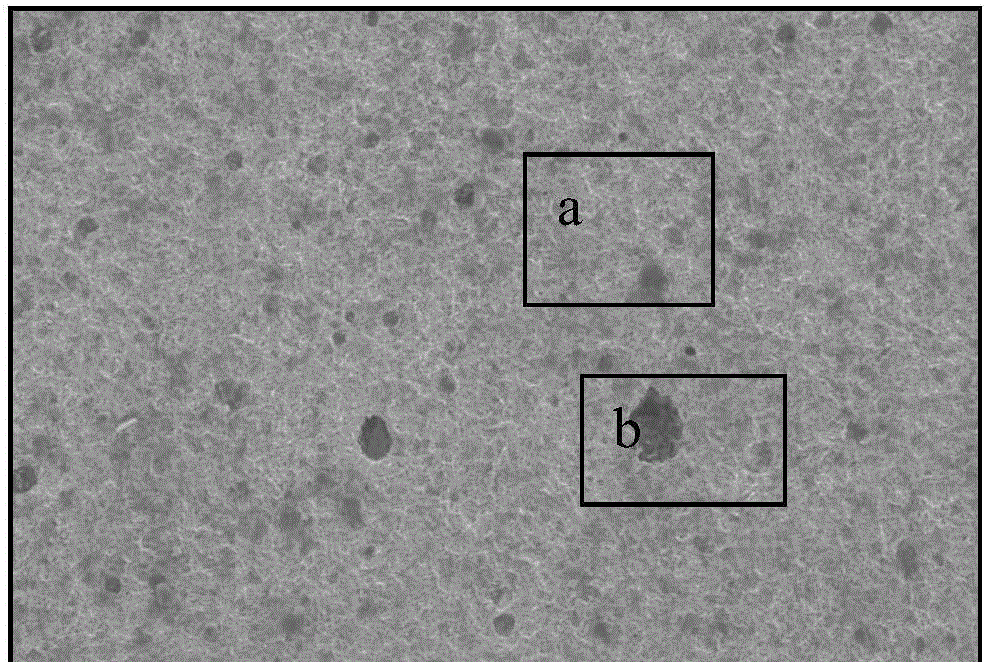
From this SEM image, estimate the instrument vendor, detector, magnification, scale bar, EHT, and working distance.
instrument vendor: Hitachi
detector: SE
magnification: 0.15 K X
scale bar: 300000 nm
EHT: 20 kV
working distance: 14.4 mm